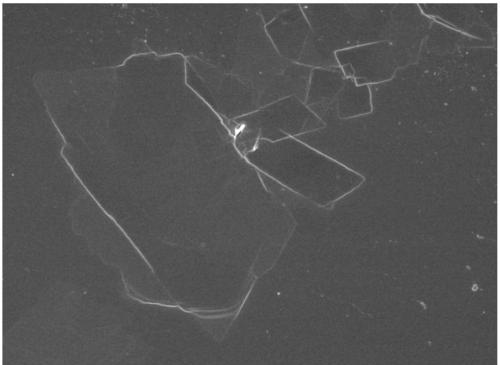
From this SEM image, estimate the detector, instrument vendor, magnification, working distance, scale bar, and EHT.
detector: SEI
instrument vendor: JEOL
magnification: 2.7 K X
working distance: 8.4 mm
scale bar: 10000 nm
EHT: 5 kV